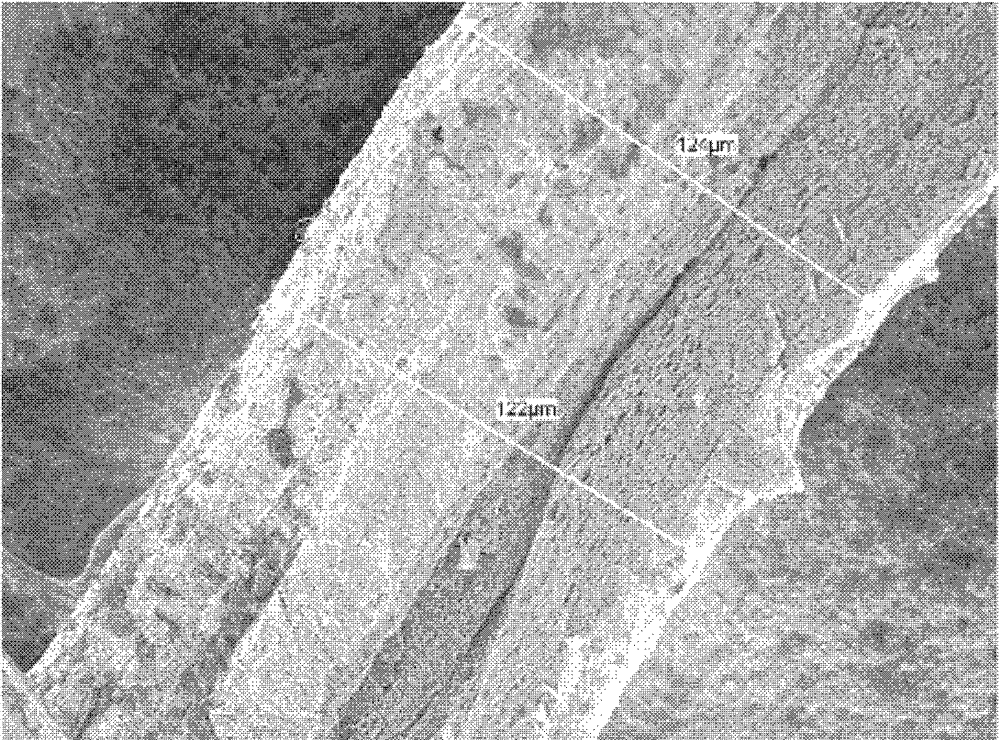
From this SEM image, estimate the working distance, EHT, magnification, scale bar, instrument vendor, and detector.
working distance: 8.5 mm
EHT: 2 kV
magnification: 0.5 K X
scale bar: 10000 nm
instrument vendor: JEOL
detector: SEI